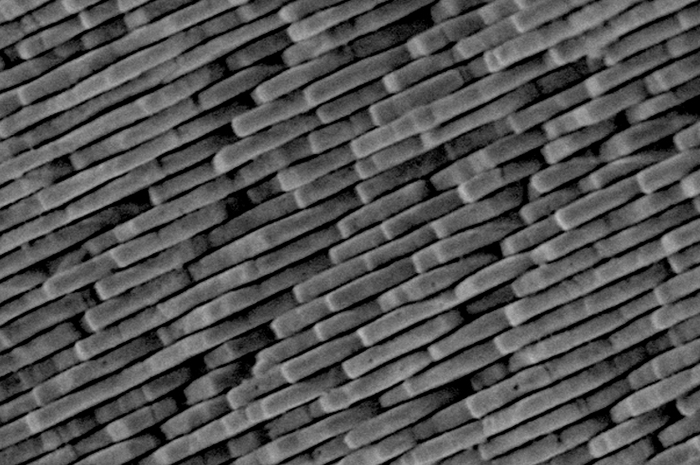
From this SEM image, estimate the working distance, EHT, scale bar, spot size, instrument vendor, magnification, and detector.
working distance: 15 mm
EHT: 5 kV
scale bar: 1000 nm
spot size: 20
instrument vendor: JEOL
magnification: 10 K X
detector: SEI